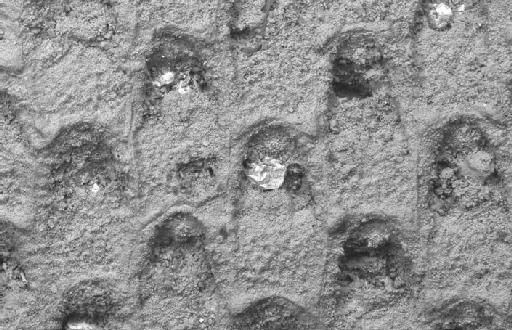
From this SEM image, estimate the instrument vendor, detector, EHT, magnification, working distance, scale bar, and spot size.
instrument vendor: Zeiss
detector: QBSD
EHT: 20 kV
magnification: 0.225 K X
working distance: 15 mm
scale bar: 100000 nm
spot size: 500